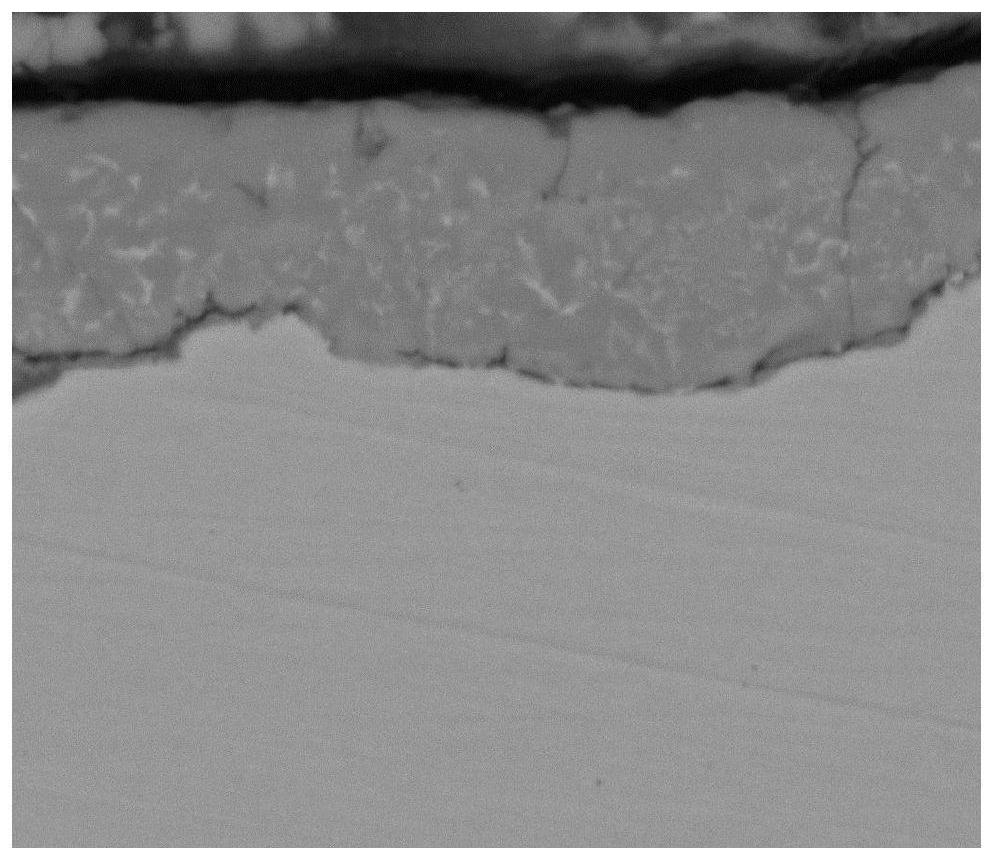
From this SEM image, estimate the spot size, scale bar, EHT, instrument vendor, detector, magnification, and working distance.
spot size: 4.5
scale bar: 10000 nm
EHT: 20 kV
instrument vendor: FEI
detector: SSD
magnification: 5 K X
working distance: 11.8 mm